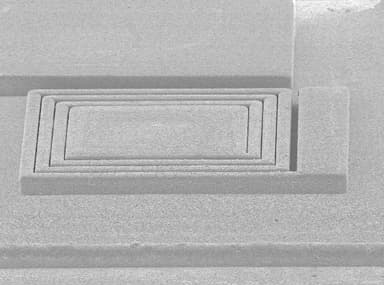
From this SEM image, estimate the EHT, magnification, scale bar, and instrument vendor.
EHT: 5 kV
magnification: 0.045 K X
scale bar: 667000 nm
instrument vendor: JEOL